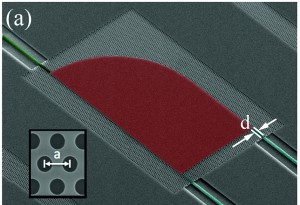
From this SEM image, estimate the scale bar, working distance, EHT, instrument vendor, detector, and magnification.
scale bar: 20000 nm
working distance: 14.3 mm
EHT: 10 kV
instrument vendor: Hitachi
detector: SE(M)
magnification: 2 K X